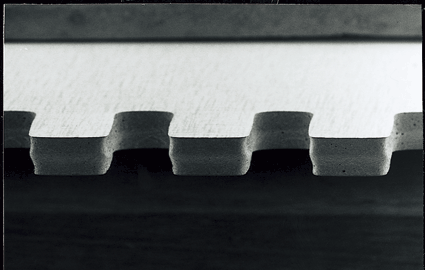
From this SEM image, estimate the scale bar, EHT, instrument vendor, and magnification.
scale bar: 100000 nm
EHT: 5 kV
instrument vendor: JEOL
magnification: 0.075 K X